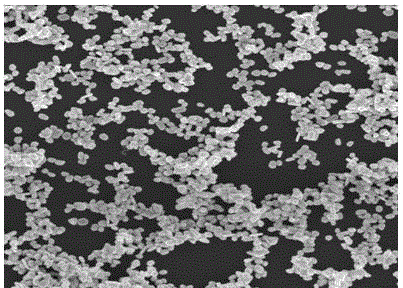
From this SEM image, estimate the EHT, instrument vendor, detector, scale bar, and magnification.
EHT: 5 kV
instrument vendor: FEI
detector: TLD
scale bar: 1000 nm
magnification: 100 K X